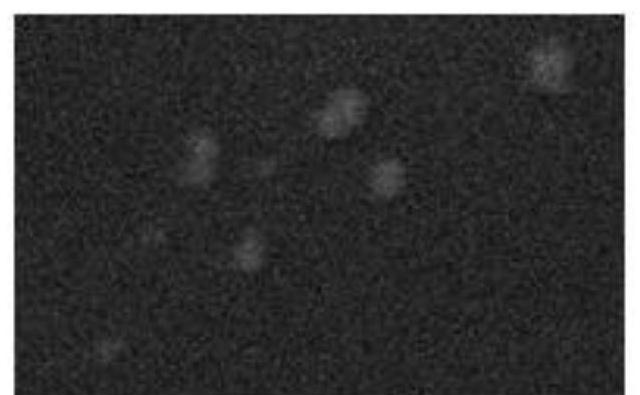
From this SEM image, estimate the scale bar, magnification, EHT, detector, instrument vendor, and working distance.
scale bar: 100 nm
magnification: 30 K X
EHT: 8 kV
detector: SEI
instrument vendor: JEOL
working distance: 7.6 mm